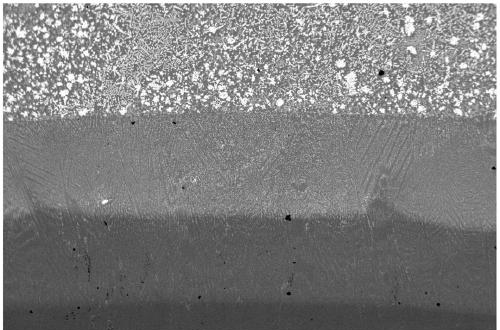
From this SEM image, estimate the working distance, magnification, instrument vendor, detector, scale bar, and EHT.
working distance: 22 mm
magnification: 0.05 K X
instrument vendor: Zeiss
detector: QBSD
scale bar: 200000 nm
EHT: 20 kV